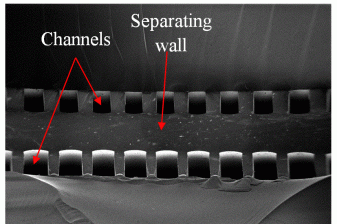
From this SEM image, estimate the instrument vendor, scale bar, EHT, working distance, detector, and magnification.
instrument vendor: Zeiss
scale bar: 200000 nm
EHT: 1.5 kV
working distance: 5.9 mm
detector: InLens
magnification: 0.147 K X